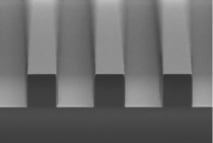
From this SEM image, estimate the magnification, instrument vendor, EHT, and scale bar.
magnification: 0.3 K X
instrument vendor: JEOL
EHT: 10 kV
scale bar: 50000 nm